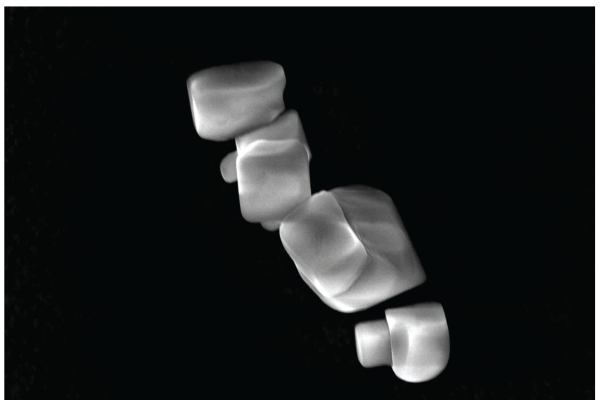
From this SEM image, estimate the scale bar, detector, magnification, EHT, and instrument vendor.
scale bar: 10000 nm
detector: SEI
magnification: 2 K X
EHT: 20 kV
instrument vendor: JEOL